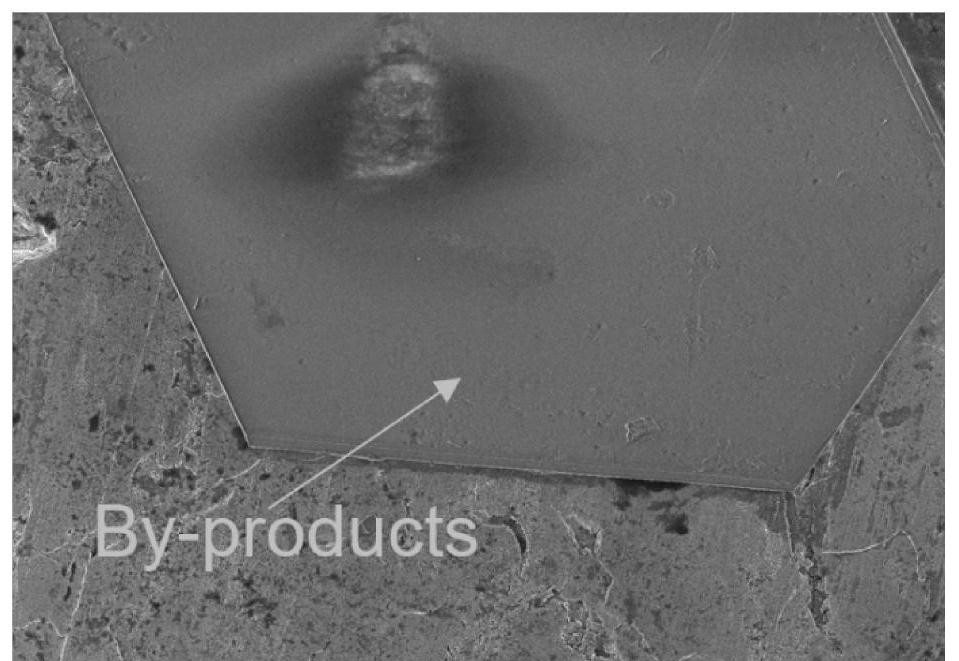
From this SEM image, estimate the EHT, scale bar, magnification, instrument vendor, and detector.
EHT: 3 kV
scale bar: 20000 nm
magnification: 2 K X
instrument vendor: Hitachi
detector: SE(UL)